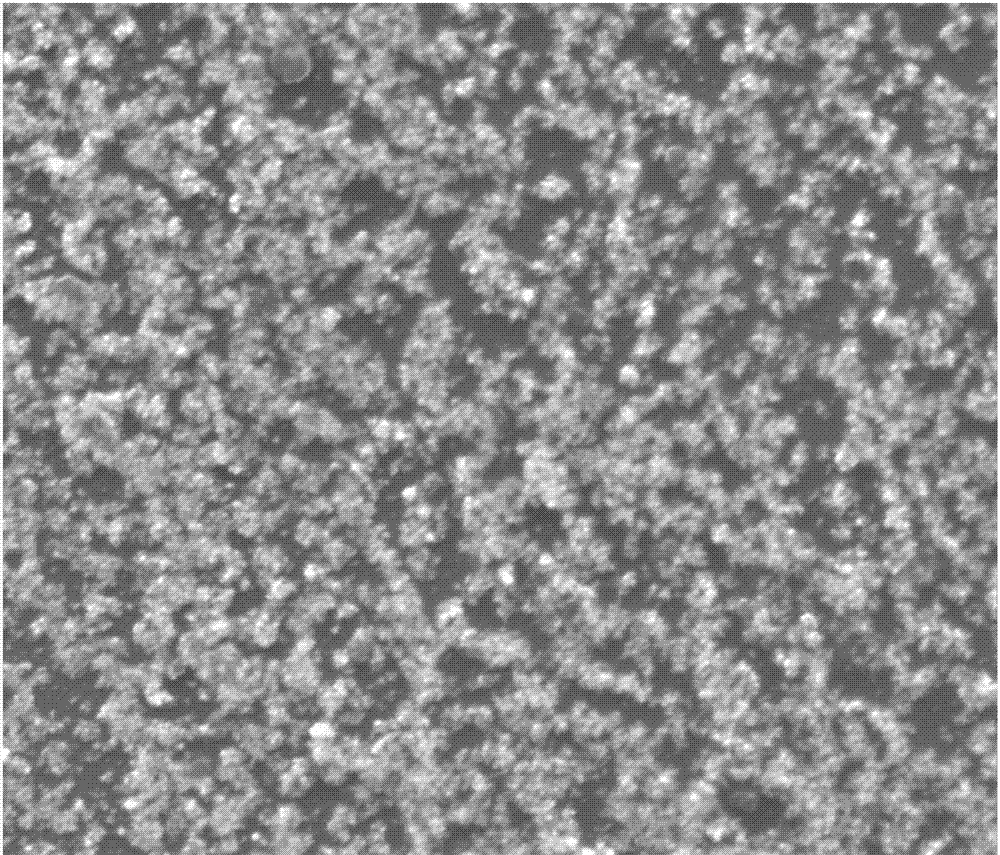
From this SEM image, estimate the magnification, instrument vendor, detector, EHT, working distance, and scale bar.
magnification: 10 K X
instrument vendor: FEI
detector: ETD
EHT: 20 kV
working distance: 11.9 mm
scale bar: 10000 nm